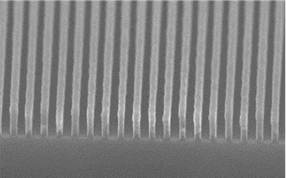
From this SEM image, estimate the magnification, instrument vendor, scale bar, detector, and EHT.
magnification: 80 K X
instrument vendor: Hitachi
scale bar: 500 nm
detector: SE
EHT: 5 kV